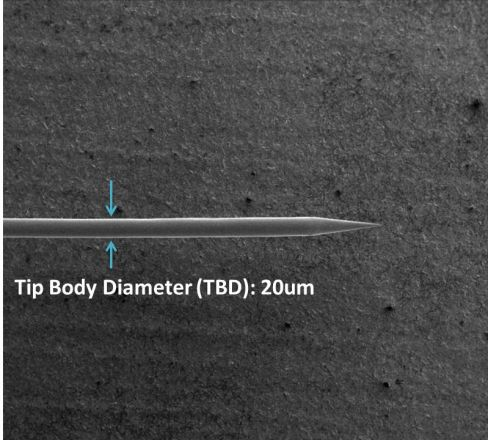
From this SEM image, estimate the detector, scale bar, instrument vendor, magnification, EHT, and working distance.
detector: ETD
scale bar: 1000000 nm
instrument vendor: FEI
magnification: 0.08 K X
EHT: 5 kV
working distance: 5 mm